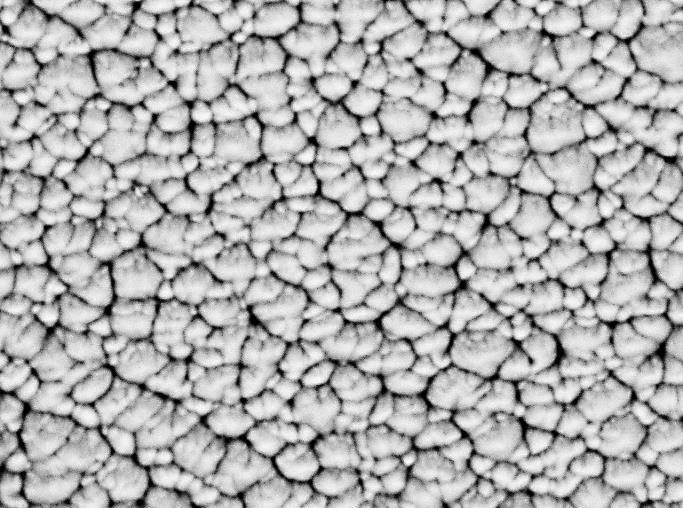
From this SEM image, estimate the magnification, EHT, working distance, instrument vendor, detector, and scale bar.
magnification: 20 K X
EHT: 5 kV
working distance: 10.1 mm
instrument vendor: JEOL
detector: LABE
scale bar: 1000 nm